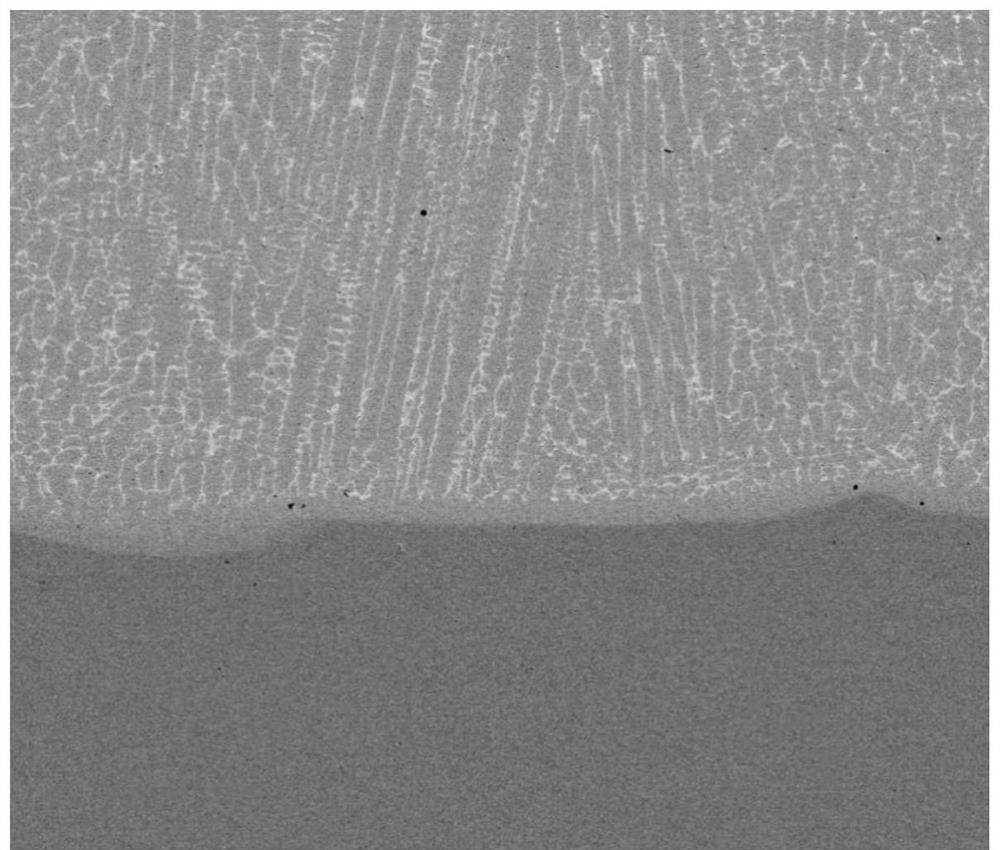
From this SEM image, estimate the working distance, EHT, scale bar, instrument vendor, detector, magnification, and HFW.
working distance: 11.1 mm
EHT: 15 kV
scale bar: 100000 nm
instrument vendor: FEI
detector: BSED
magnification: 1 K X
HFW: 298 µm